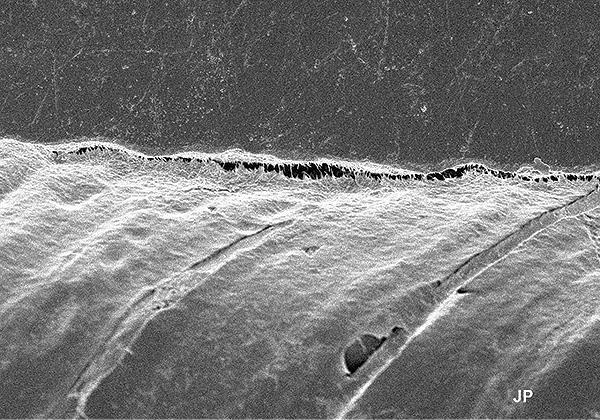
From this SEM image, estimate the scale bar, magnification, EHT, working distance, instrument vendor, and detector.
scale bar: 20000 nm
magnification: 2.5 K X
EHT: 4 kV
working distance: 11.9 mm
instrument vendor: Hitachi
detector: SE(M)